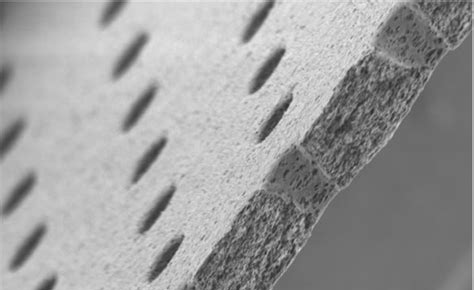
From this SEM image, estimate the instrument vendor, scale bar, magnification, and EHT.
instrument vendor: JEOL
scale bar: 10000 nm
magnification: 1 K X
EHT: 5 kV